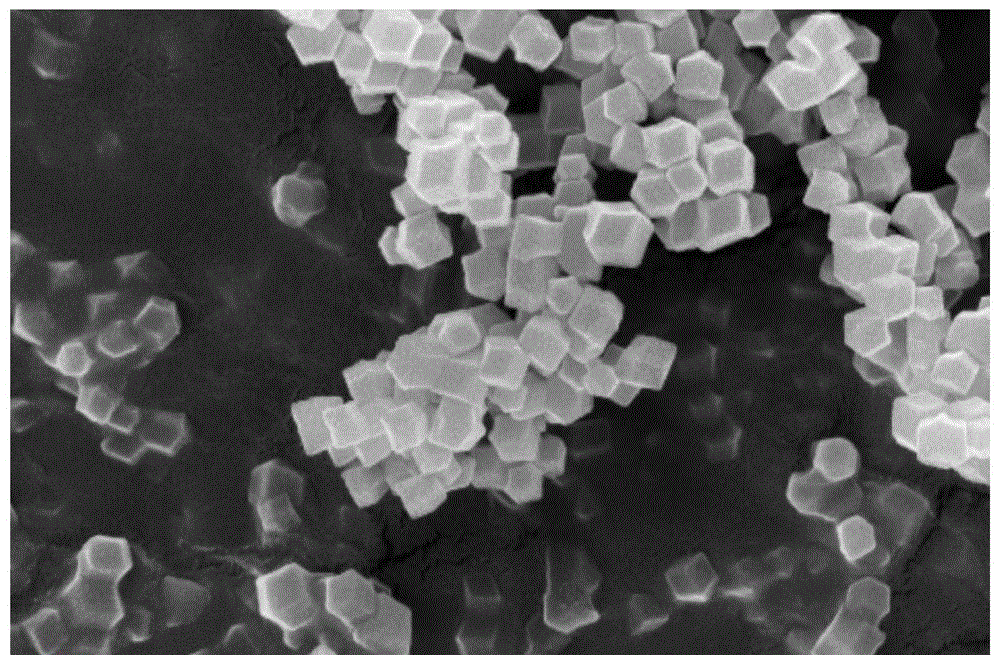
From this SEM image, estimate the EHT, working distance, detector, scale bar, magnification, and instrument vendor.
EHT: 5 kV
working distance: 4 mm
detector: TLD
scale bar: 1000 nm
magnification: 50 K X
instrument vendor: FEI